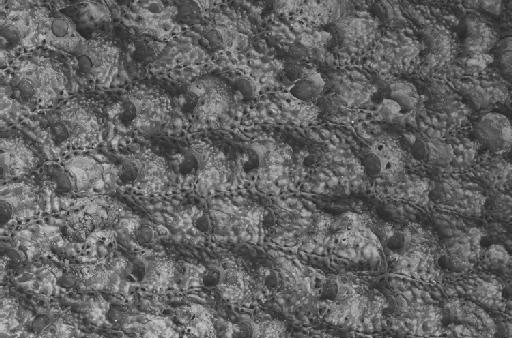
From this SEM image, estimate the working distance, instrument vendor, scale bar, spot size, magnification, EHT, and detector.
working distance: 17 mm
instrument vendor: Zeiss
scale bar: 100000 nm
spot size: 500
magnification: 0.1 K X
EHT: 15 kV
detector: BSD